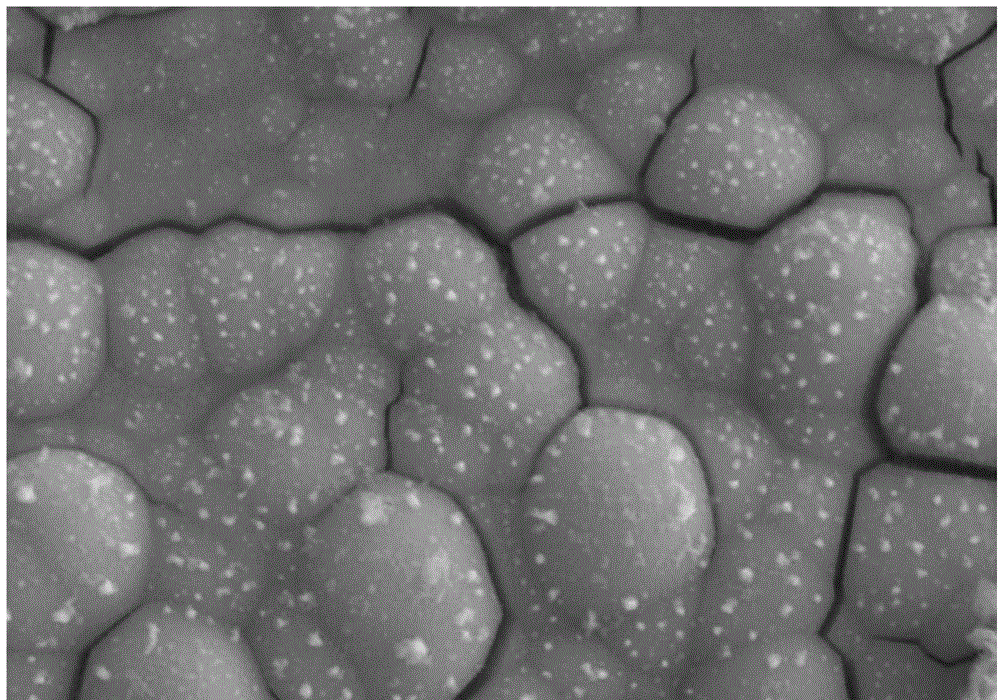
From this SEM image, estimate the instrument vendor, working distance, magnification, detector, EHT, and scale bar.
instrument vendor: Zeiss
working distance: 7.9 mm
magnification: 10 K X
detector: SE2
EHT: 15 kV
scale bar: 1000 nm